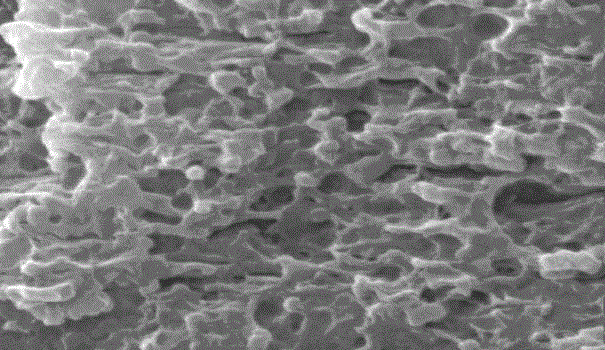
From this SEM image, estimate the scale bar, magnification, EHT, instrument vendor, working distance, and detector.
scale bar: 2000 nm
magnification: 10 K X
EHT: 20 kV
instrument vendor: FEI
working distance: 12.7 mm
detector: SE